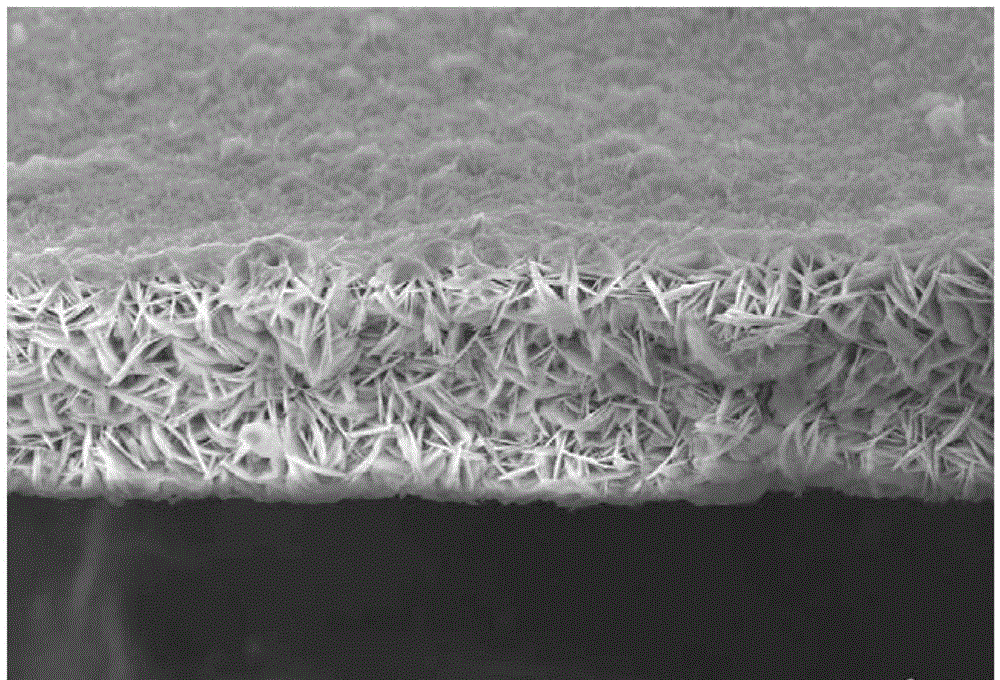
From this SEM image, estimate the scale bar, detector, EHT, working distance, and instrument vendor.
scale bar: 10000 nm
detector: SE2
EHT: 15 kV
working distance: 10.6 mm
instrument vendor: Zeiss